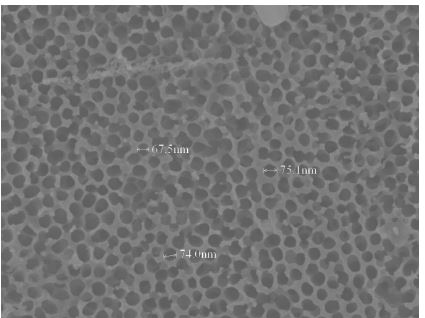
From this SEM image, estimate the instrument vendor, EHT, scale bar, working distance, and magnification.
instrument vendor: JEOL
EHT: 10 kV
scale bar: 100 nm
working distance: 6.3 mm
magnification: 50 K X